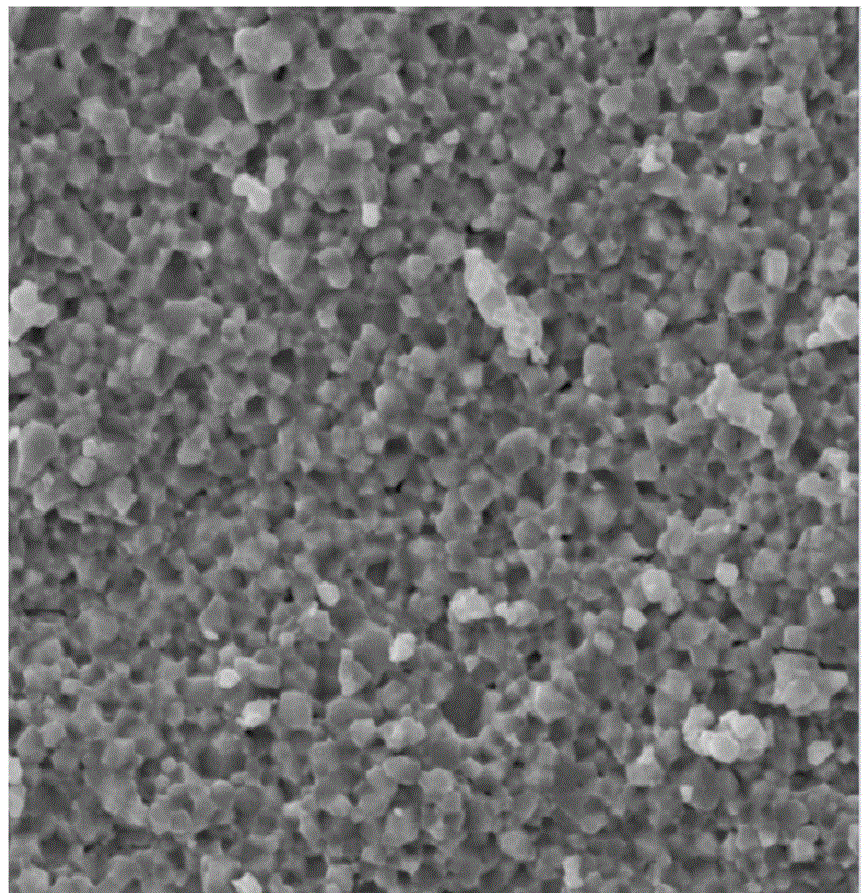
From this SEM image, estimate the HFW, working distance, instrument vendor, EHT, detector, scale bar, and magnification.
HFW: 44.9 µm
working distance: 14.98 mm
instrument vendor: Tescan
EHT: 20 kV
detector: SE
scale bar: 10000 nm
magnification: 5 K X